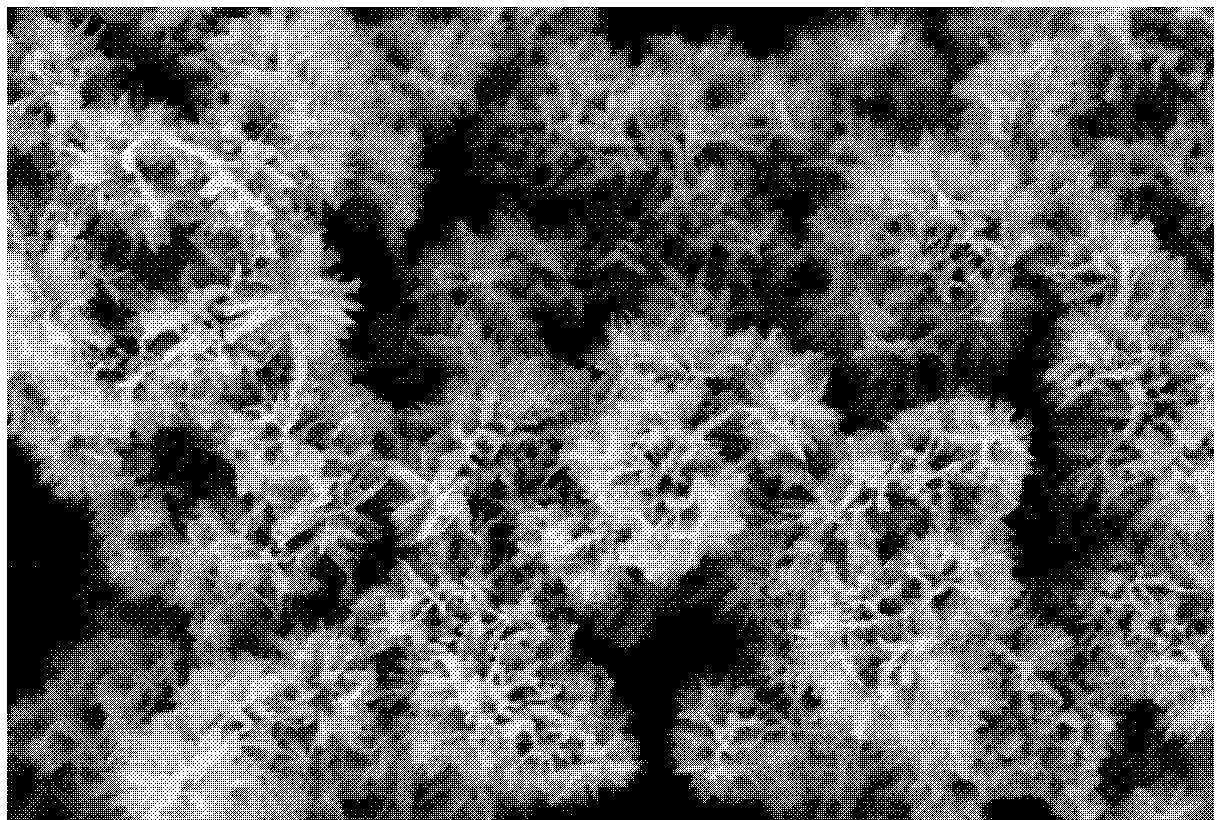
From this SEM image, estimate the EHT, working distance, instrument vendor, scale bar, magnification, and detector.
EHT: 3 kV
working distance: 8.2 mm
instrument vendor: Hitachi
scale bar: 2000 nm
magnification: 25 K X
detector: SE(M,LA0)